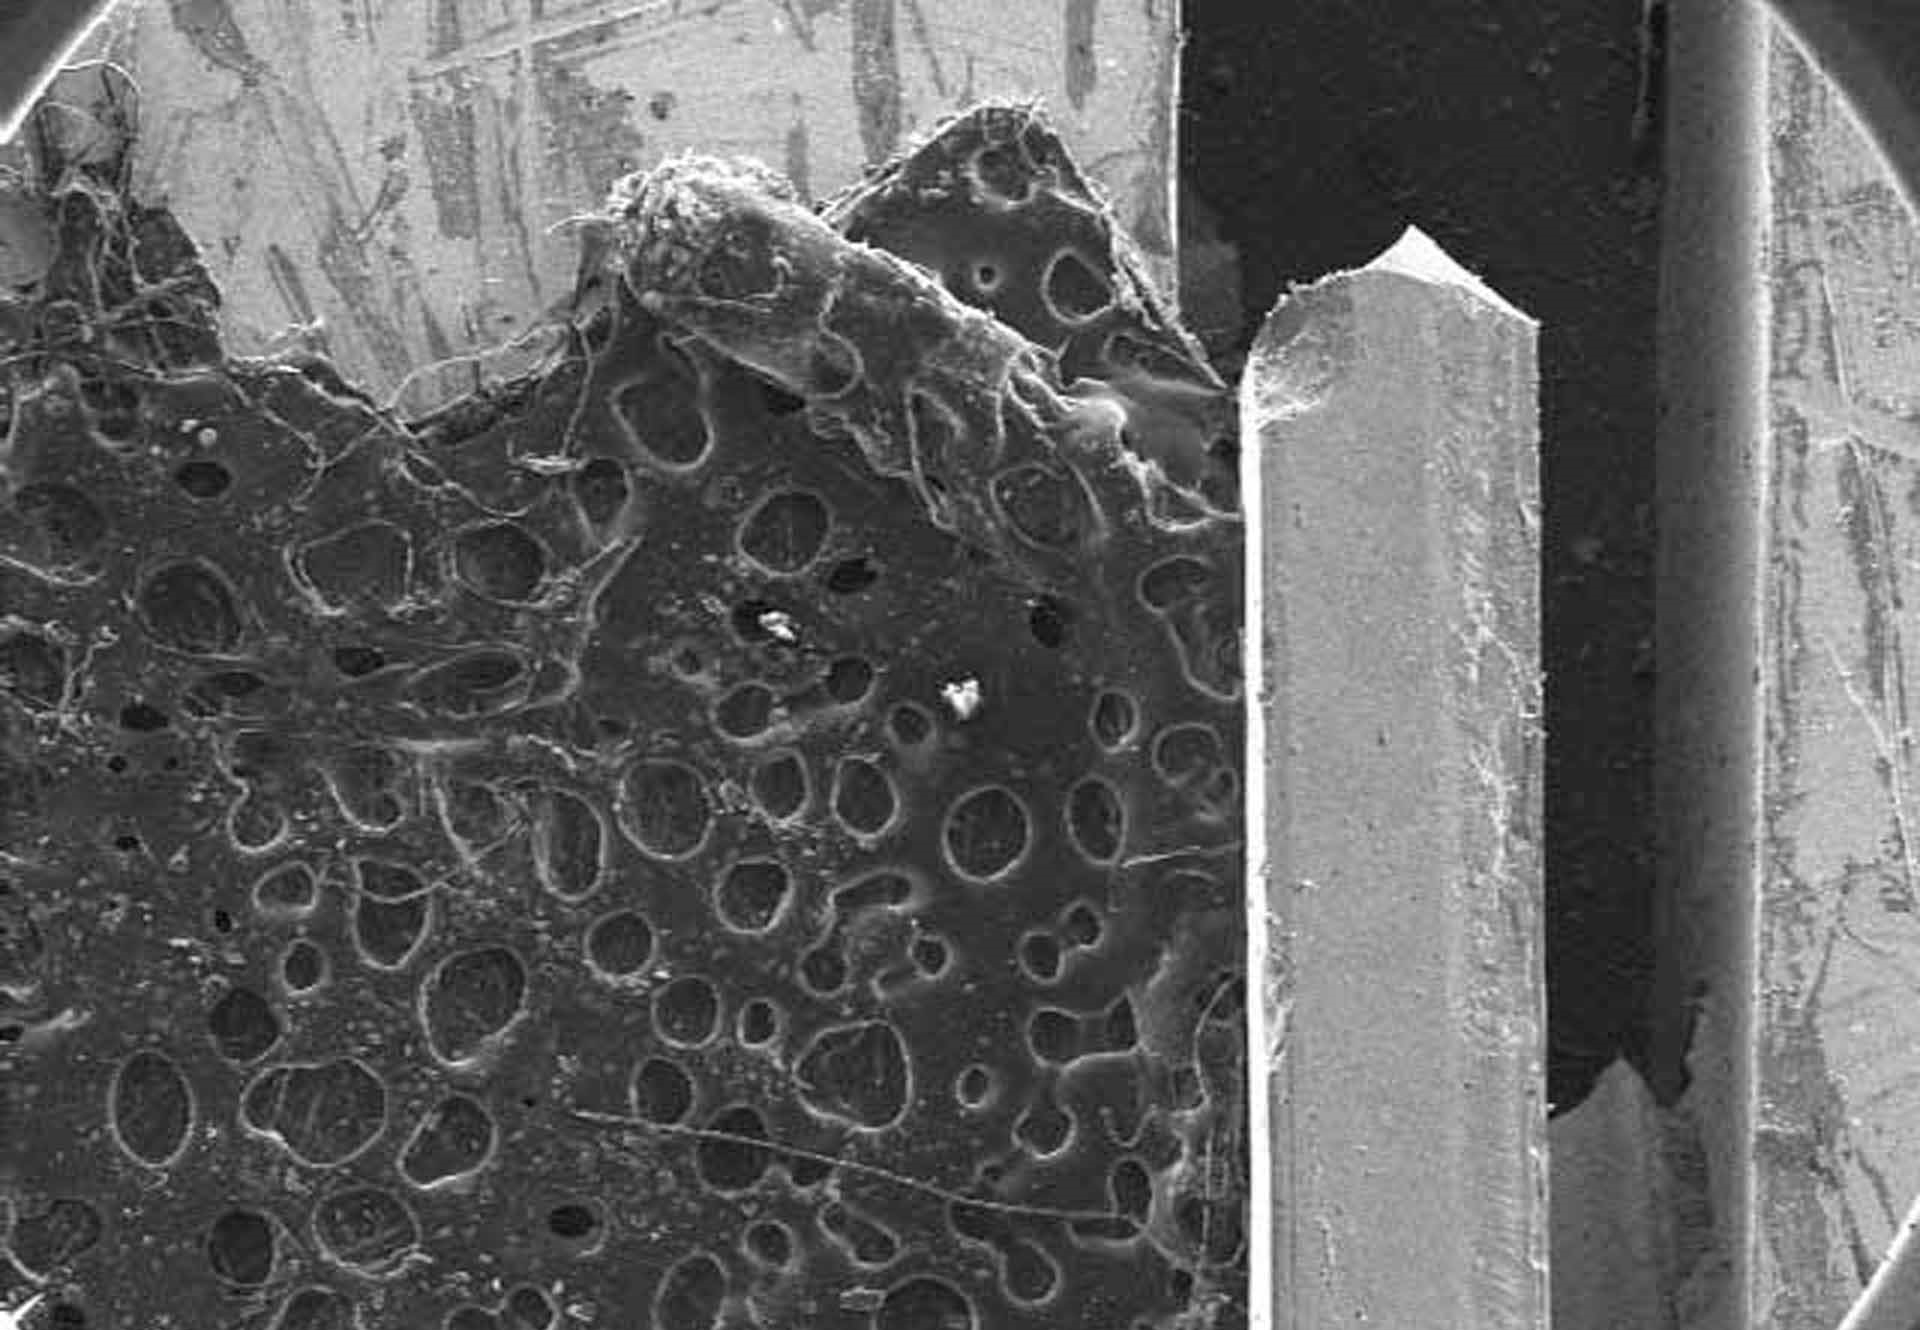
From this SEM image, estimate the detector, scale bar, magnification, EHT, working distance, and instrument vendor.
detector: SE(M)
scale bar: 2000000 nm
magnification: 0.025 K X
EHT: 15 kV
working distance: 16.6 mm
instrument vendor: Hitachi